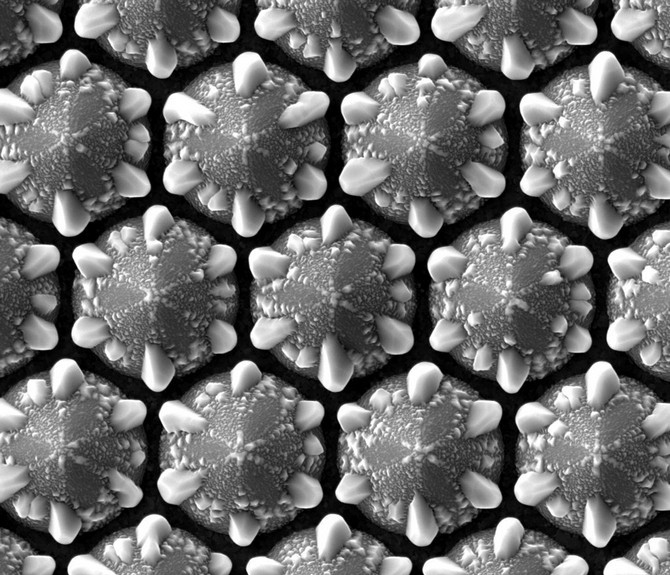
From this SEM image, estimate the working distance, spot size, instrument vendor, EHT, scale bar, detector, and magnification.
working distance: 4.9 mm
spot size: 4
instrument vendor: FEI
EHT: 10 kV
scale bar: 5000 nm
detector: ETD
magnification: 20 K X